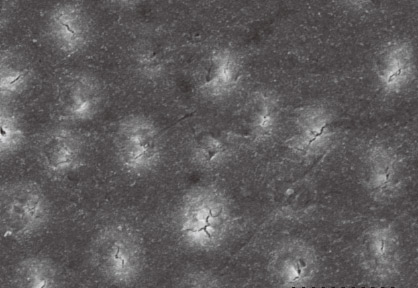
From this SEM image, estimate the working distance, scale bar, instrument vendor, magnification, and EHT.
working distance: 25.7 mm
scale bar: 10000 nm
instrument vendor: JEOL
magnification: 3 K X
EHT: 15 kV